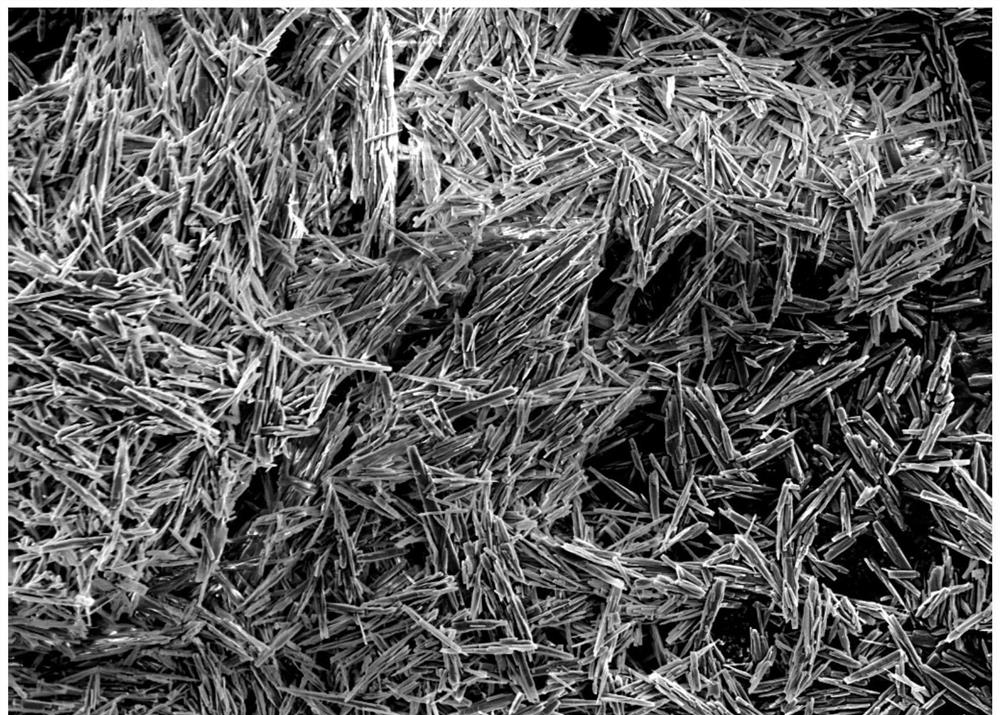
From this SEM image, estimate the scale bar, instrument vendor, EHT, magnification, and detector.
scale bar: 10000 nm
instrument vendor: Hitachi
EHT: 5 kV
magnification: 3 K X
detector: SE